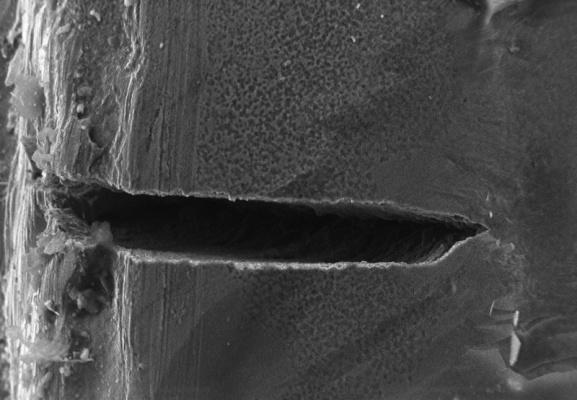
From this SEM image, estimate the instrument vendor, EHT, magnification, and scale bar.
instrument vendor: other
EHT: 27.8 kV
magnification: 0.341 K X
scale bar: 100000 nm